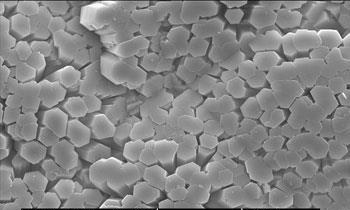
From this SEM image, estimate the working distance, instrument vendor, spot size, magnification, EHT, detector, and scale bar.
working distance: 5.7 mm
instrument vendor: FEI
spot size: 3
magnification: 3.003 K X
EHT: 15 kV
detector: SE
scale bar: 10000 nm